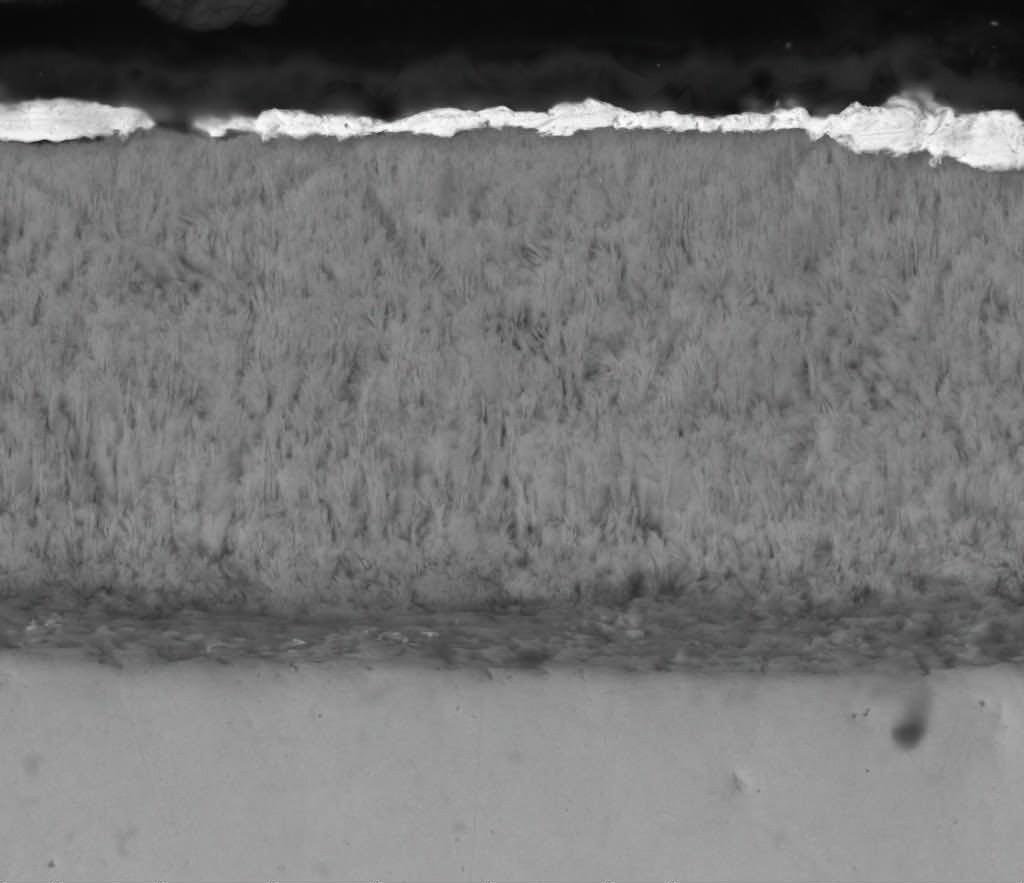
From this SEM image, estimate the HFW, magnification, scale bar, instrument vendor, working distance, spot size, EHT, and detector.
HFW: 29.8 µm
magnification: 10 K X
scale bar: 10000 nm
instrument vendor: FEI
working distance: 7.1 mm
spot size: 4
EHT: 15 kV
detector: DualBSD